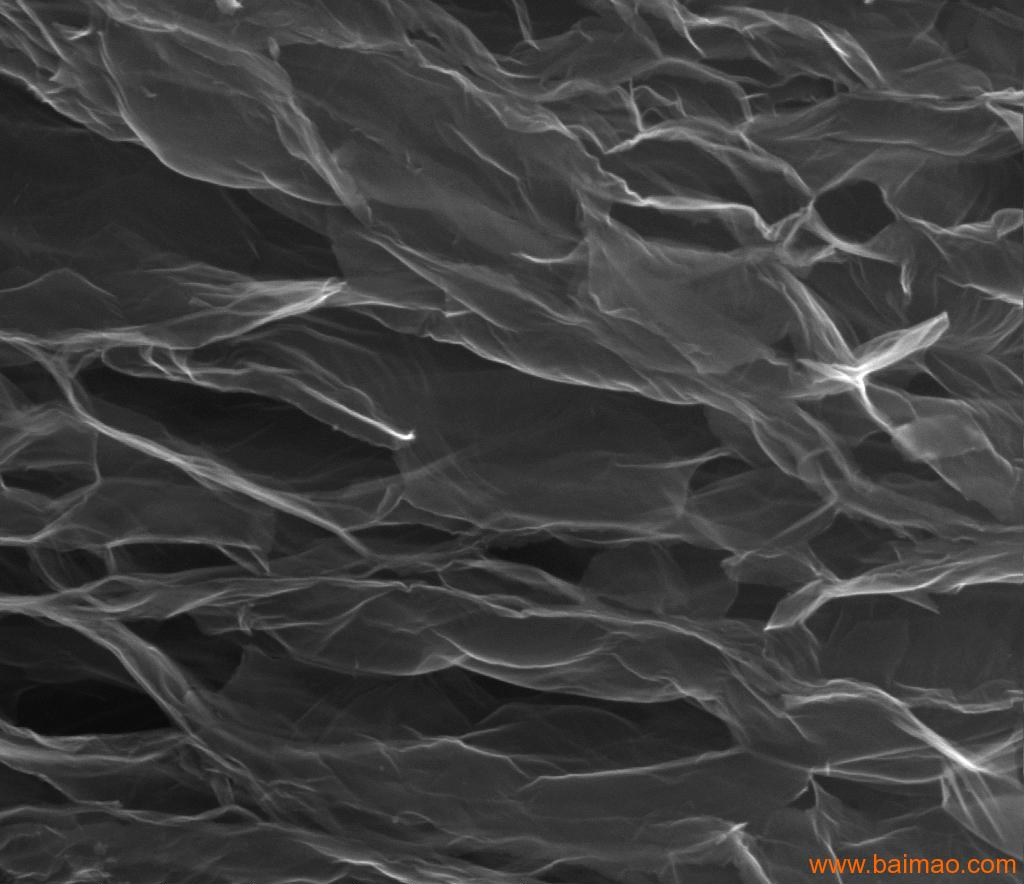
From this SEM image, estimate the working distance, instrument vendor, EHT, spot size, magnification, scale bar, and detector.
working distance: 7.9 mm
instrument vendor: FEI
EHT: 20 kV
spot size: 4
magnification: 50 K X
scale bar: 2000 nm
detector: ETD